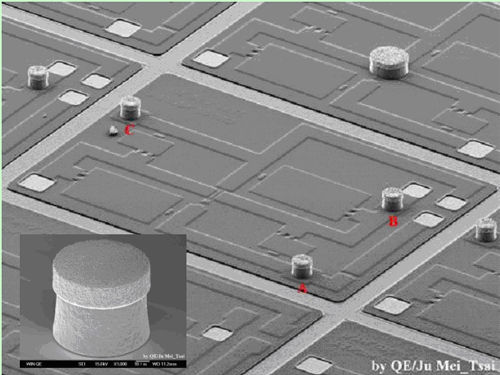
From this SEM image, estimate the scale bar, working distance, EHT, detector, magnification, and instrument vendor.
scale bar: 100000 nm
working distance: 9 mm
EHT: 15 kV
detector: LEI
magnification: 0.07 K X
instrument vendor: JEOL